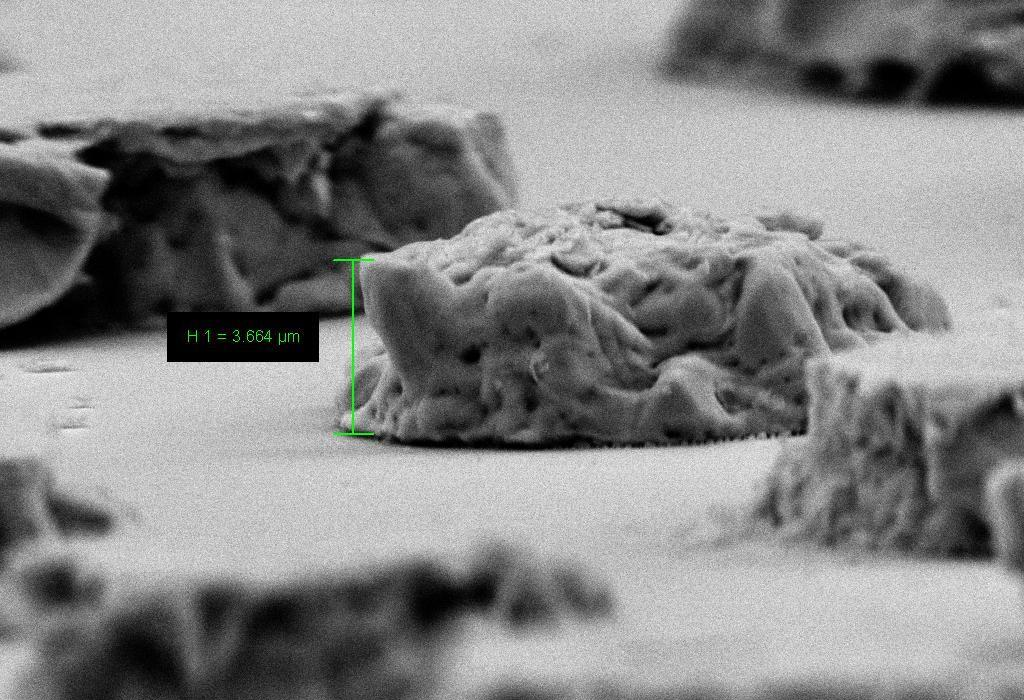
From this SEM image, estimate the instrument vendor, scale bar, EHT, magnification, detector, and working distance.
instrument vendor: Zeiss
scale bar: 2000 nm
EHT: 10 kV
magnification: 5.3 K X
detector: SE2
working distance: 9 mm